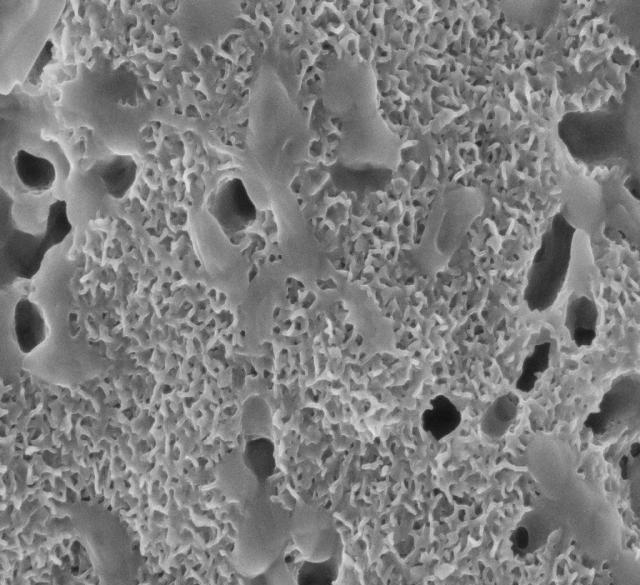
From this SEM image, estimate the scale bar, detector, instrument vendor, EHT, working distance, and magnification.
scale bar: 1000 nm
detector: InLens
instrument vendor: Zeiss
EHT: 5 kV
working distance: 3.4 mm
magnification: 30 K X